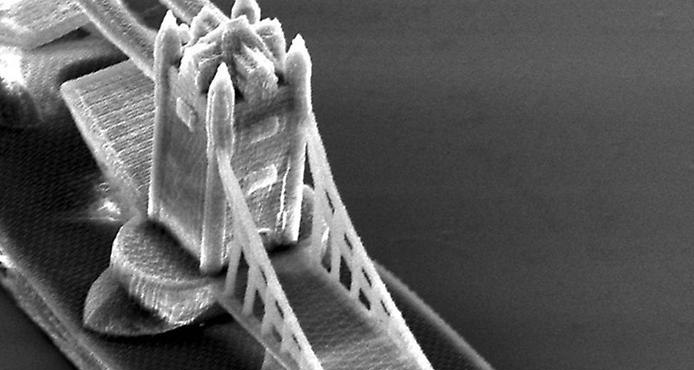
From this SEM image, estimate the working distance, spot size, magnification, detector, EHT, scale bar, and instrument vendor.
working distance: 27.1 mm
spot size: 4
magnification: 0.969 K X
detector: SE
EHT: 15 kV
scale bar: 20000 nm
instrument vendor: FEI